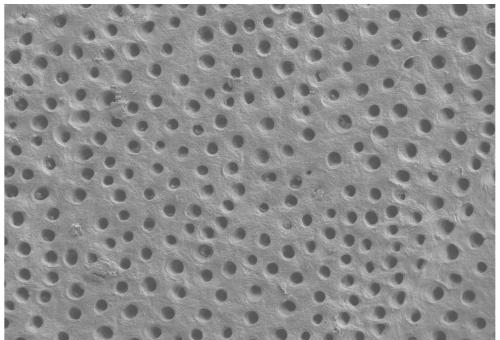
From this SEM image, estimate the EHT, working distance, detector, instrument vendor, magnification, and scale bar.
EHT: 3 kV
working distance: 5.3 mm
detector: SE2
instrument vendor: Zeiss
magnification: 1 K X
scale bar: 10000 nm